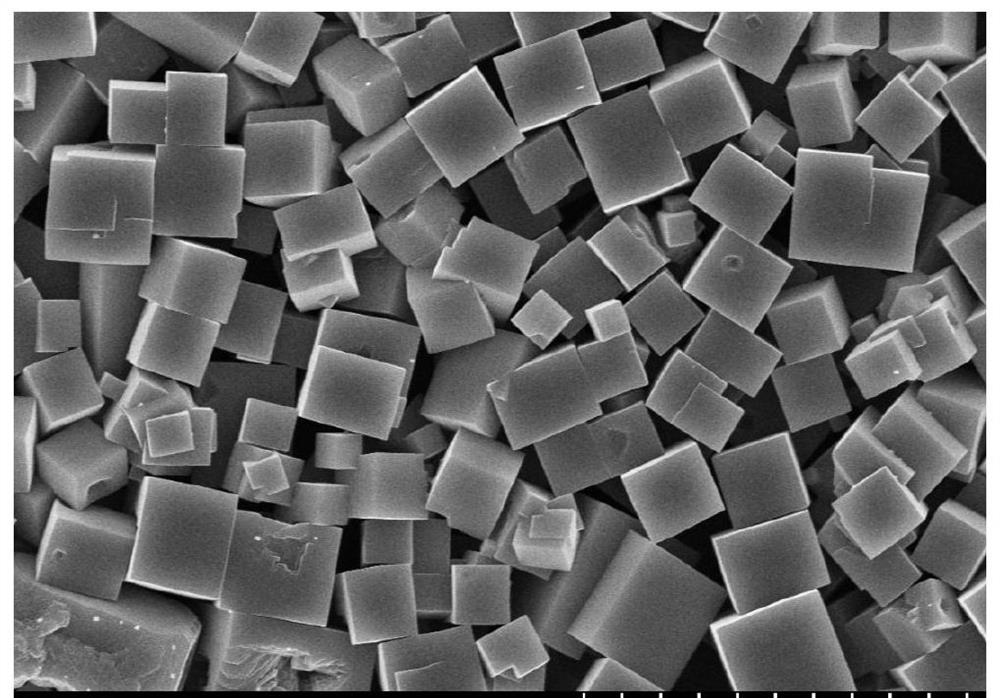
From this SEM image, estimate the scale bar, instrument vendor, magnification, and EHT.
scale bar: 10000 nm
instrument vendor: Hitachi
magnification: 5 K X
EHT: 10 kV